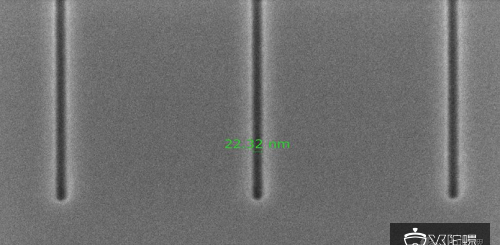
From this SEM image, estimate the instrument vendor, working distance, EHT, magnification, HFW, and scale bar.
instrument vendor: FEI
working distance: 2.1827 mm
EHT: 2 kV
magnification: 100 K X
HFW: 1.27 µm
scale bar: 500 nm